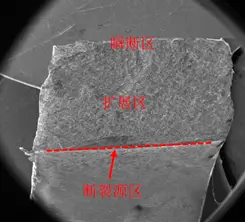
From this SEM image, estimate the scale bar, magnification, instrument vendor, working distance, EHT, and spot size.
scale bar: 2000000 nm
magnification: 0.038 K X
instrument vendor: FEI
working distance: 14.8 mm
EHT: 20 kV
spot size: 6